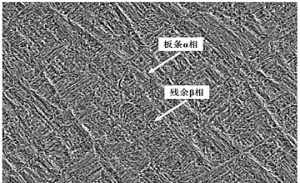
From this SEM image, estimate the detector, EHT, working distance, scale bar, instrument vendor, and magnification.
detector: SE(M)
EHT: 15 kV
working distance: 16.2 mm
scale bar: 50000 nm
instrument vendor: Hitachi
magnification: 1 K X